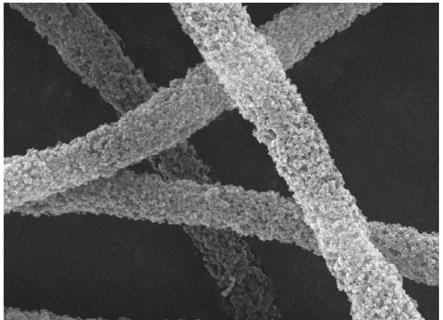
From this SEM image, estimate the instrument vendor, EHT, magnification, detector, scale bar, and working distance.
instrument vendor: JEOL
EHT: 15 kV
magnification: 8.5 K X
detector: SEI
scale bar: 1000 nm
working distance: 9 mm